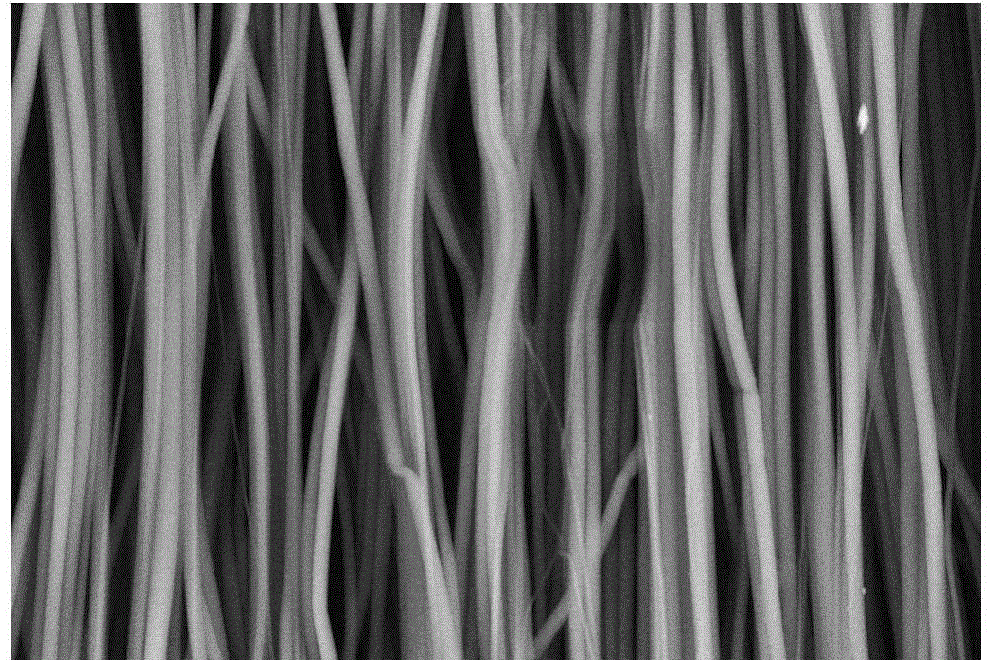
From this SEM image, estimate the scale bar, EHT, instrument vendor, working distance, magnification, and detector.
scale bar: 10000 nm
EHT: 15 kV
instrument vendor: Zeiss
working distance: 9.9 mm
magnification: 1 K X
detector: RBSD